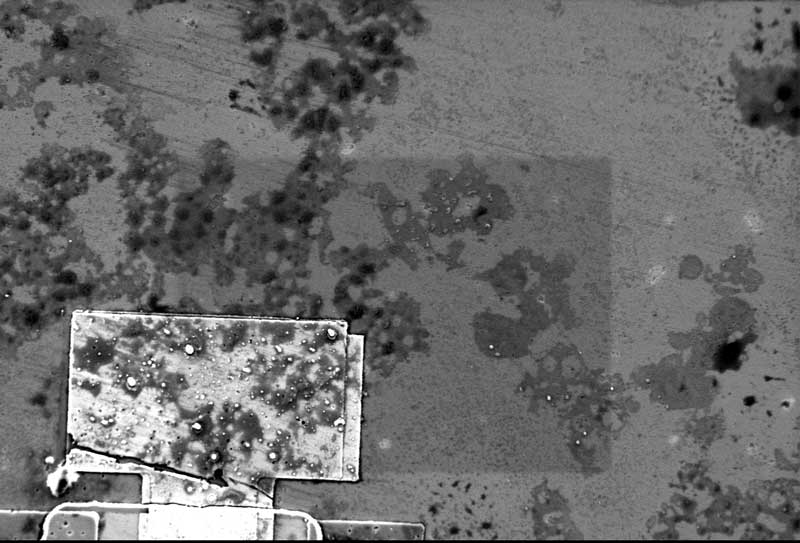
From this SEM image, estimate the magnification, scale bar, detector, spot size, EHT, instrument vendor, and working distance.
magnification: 0.5 K X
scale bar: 100000 nm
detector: SE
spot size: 14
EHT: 20 kV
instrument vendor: other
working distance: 7.5 mm